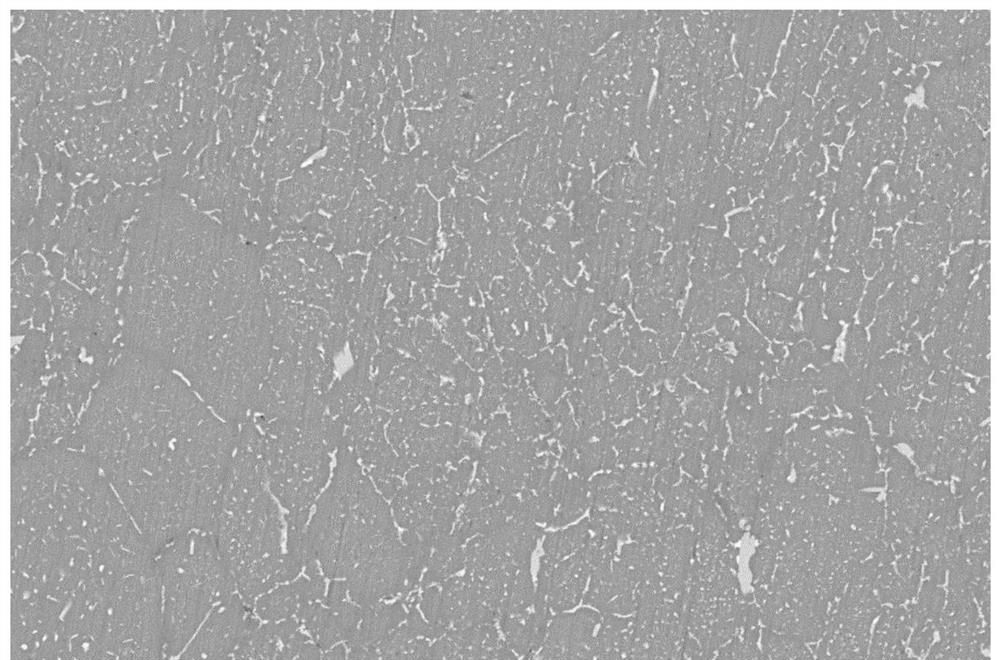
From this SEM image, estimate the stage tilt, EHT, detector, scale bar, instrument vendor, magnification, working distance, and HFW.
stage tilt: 0°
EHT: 15 kV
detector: CBS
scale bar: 40000 nm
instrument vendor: FEI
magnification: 2 K X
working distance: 5.7 mm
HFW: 207 µm